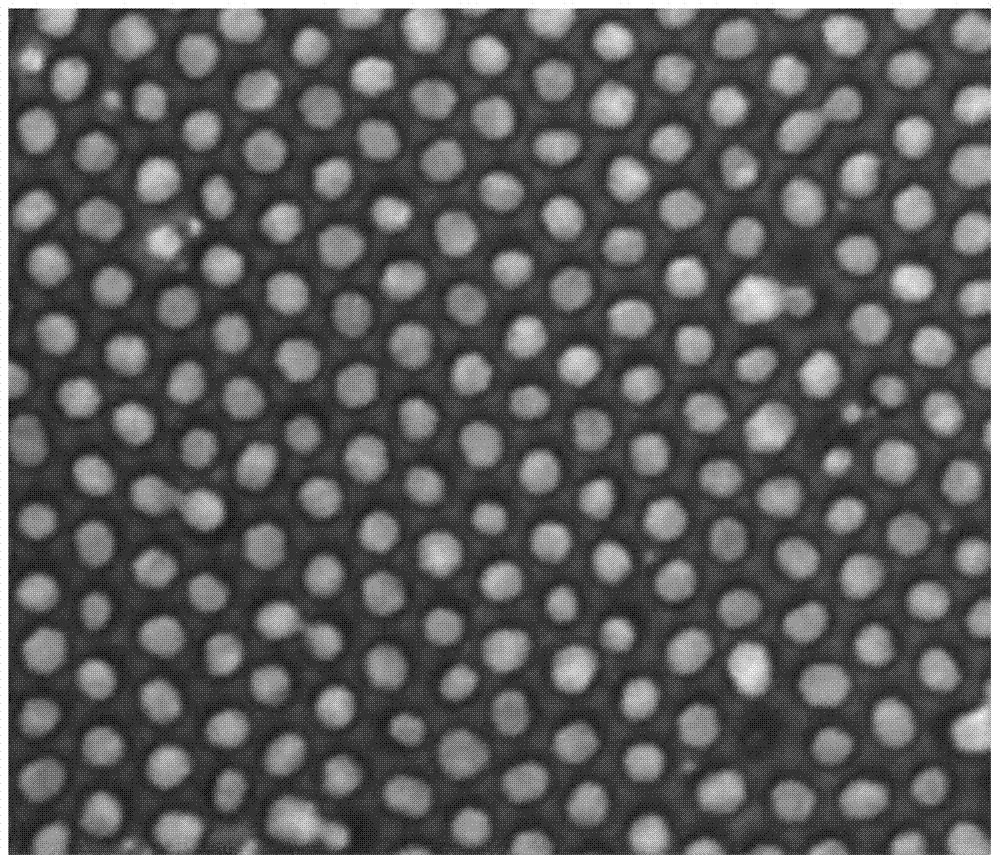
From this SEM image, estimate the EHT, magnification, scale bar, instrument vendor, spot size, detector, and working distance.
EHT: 20 kV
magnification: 200 K X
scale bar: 500 nm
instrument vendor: FEI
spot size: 3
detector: ETD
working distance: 10.1 mm